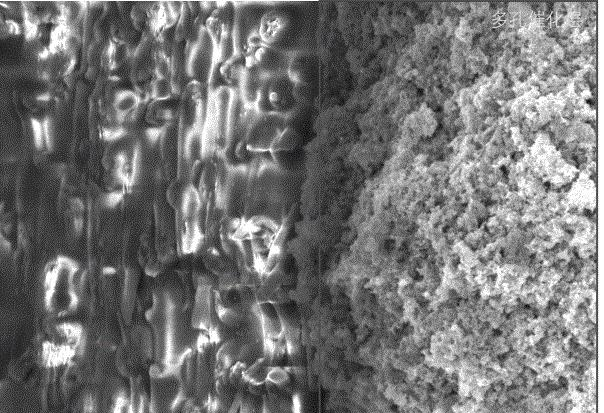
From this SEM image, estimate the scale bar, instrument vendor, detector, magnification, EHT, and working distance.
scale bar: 2000 nm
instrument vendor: Zeiss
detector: InlensDuo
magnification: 19.42 K X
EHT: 5 kV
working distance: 12 mm